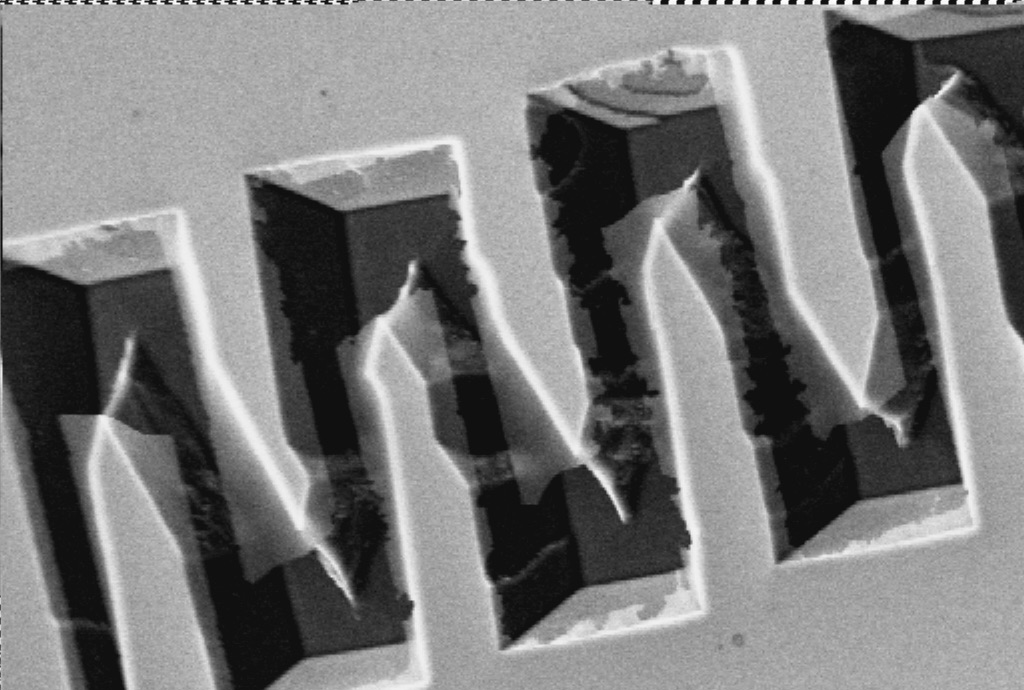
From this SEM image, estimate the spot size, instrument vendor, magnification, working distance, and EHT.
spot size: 10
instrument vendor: other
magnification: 0.23 K X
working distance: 20 mm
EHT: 30 kV